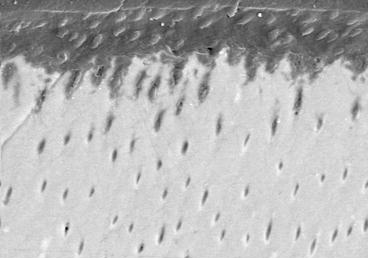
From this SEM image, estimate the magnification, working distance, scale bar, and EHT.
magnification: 2.059 K X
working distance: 27.3 mm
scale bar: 20000 nm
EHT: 30 kV